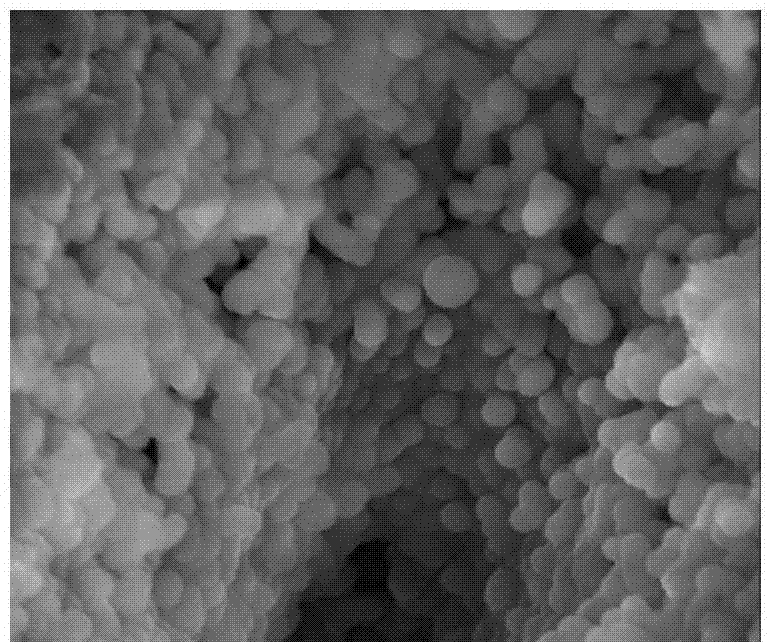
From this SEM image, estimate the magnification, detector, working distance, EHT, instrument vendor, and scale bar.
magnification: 50 K X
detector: TLD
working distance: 5.3 mm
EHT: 10 kV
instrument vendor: FEI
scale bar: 1000 nm